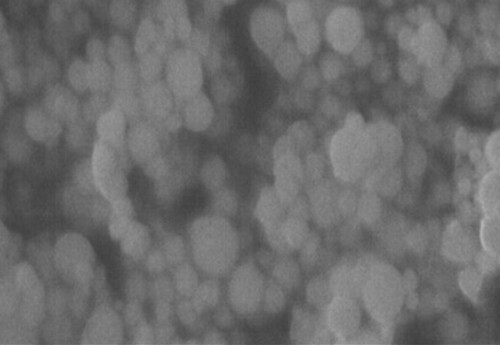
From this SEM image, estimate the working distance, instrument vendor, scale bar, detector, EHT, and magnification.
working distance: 9.7 mm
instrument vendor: FEI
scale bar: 200 nm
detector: ETD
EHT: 20 kV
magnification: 10 K X